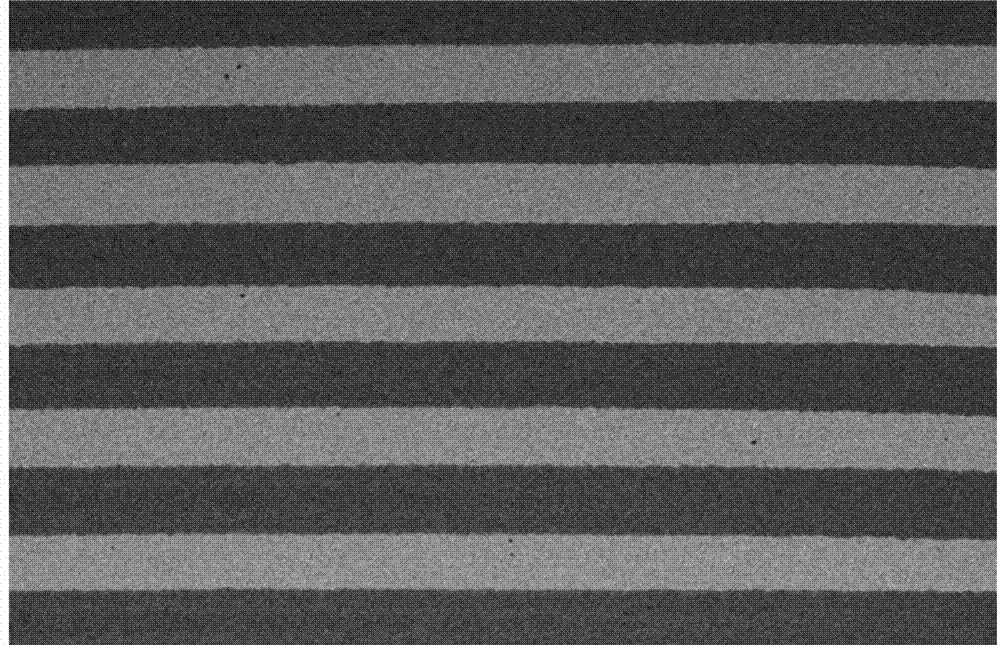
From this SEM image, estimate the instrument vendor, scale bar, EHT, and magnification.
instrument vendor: JEOL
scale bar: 1000000 nm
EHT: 20 kV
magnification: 0.017 K X